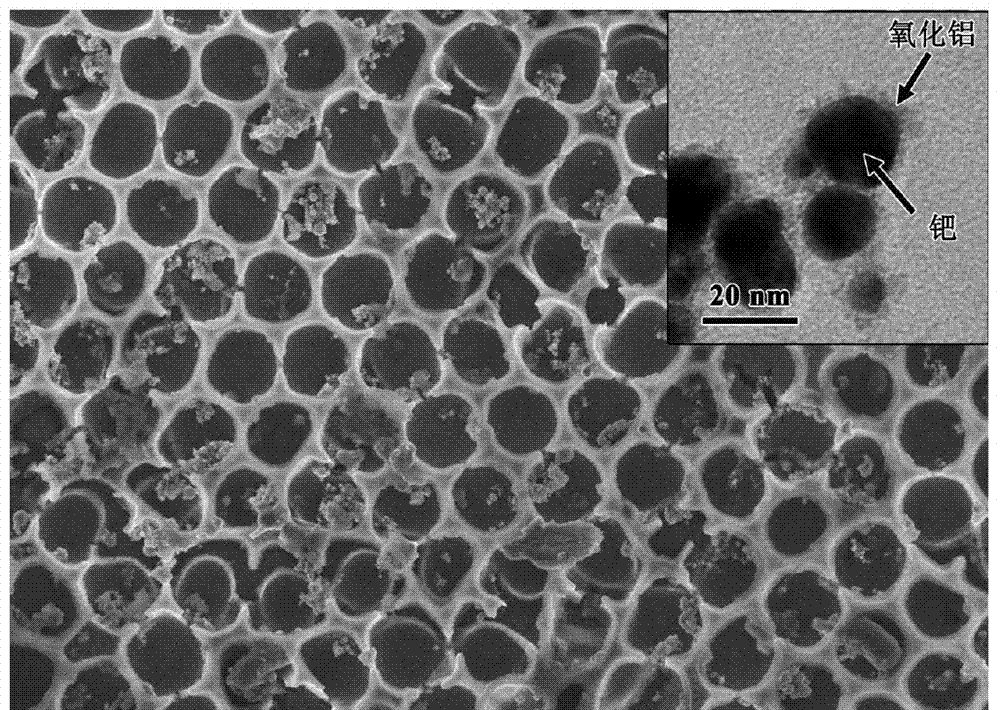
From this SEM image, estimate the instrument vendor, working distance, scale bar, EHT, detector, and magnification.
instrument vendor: Hitachi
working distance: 11.1 mm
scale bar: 5000 nm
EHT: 5 kV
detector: SE(U)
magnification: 10 K X